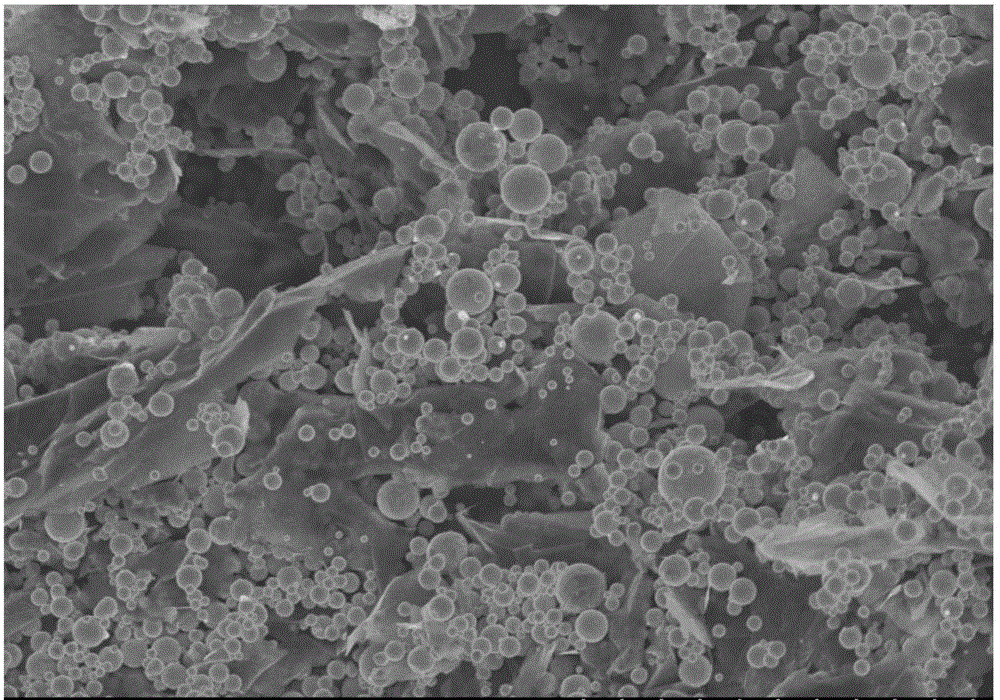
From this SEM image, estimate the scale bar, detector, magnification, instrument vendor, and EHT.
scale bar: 10000 nm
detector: SE(U)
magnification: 5 K X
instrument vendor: Hitachi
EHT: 4 kV